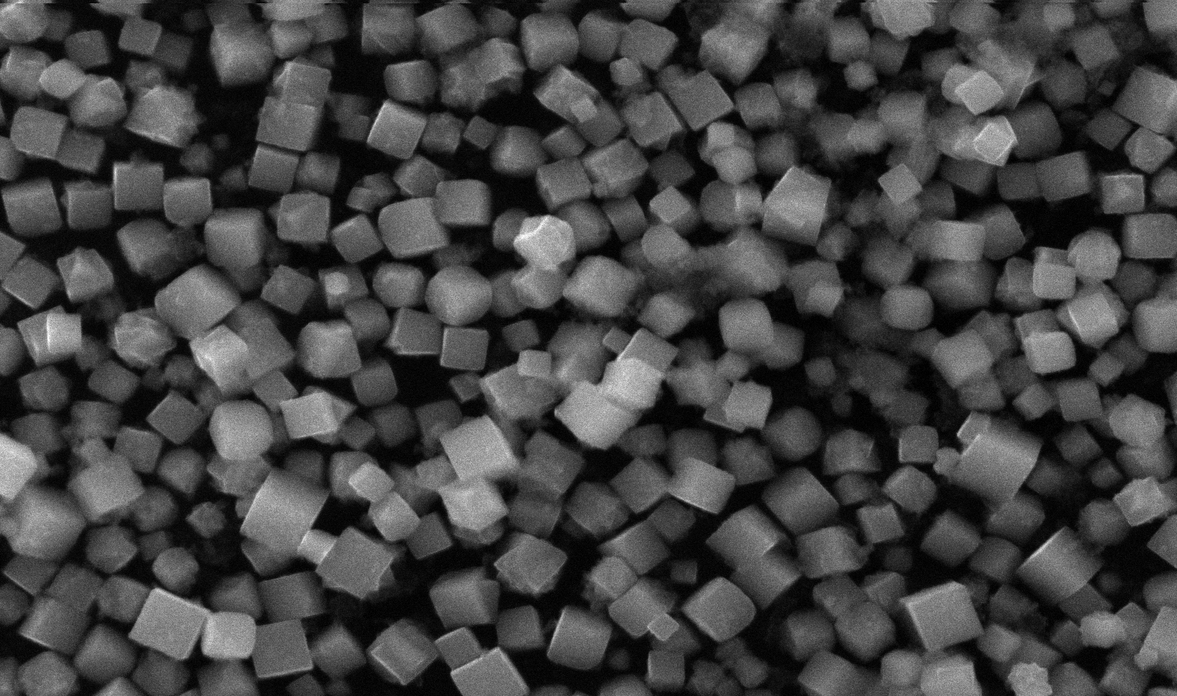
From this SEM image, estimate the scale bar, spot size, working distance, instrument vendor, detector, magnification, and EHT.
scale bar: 5000 nm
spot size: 2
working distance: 10 mm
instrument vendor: FEI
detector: SE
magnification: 8.89 K X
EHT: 30 kV